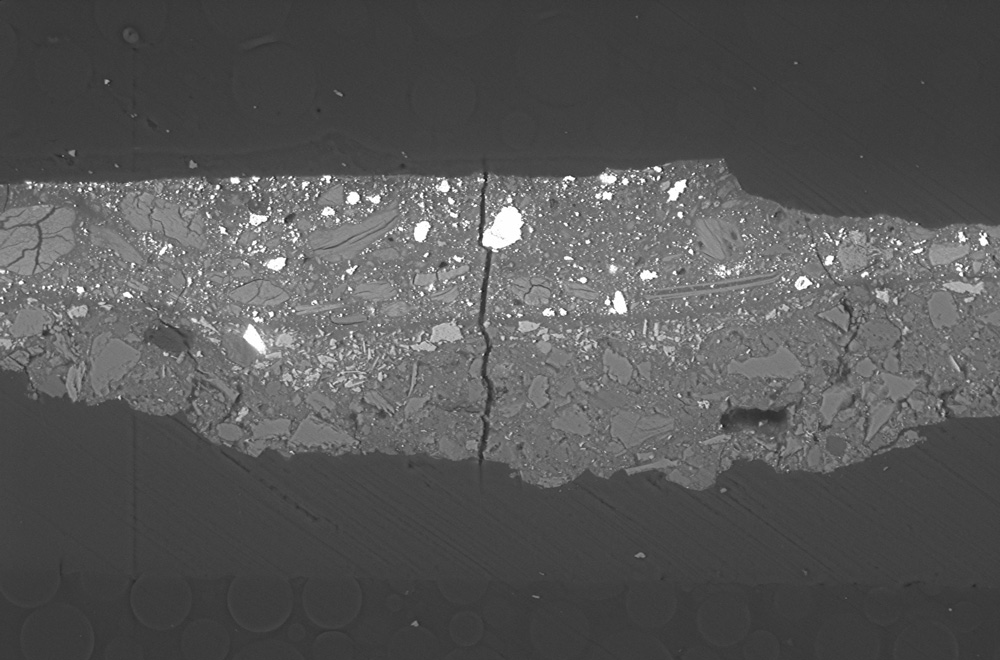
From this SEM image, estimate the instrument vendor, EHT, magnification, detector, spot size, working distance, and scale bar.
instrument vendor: Zeiss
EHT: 20 kV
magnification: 0.5 K X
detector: NTS BSD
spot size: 410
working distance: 8 mm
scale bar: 20000 nm